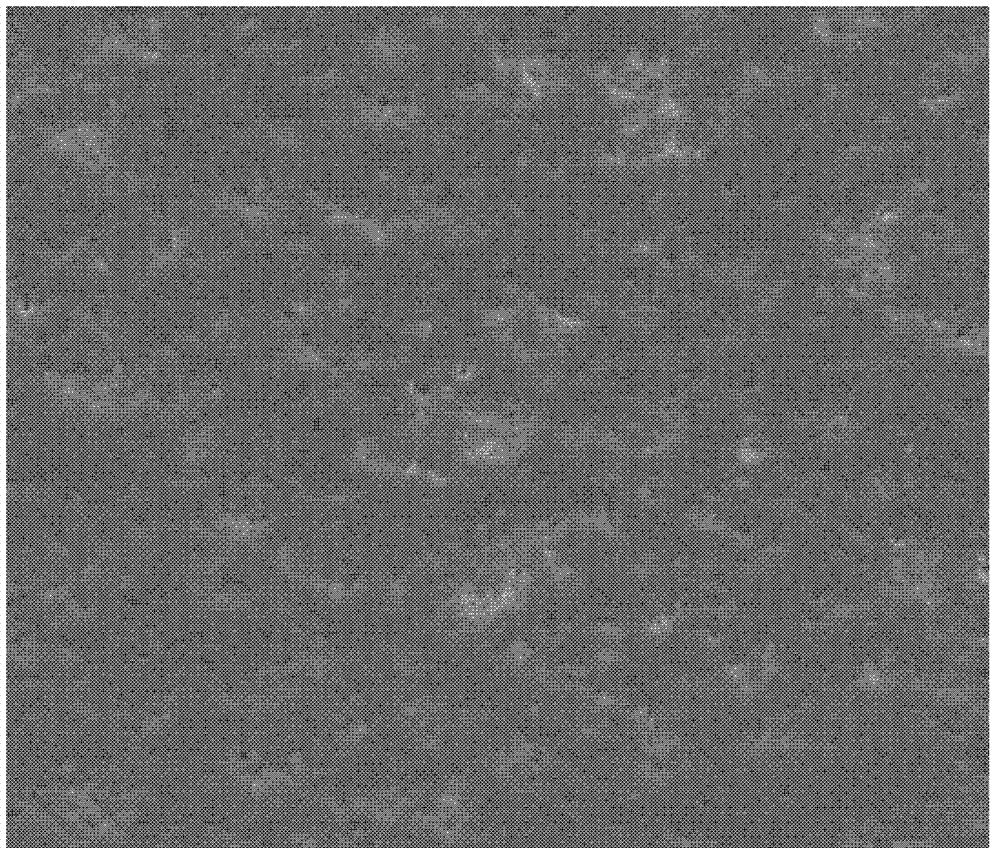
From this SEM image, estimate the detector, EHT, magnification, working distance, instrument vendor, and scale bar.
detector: SE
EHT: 20 kV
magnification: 2 K X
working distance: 13.6 mm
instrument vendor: FEI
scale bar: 50000 nm